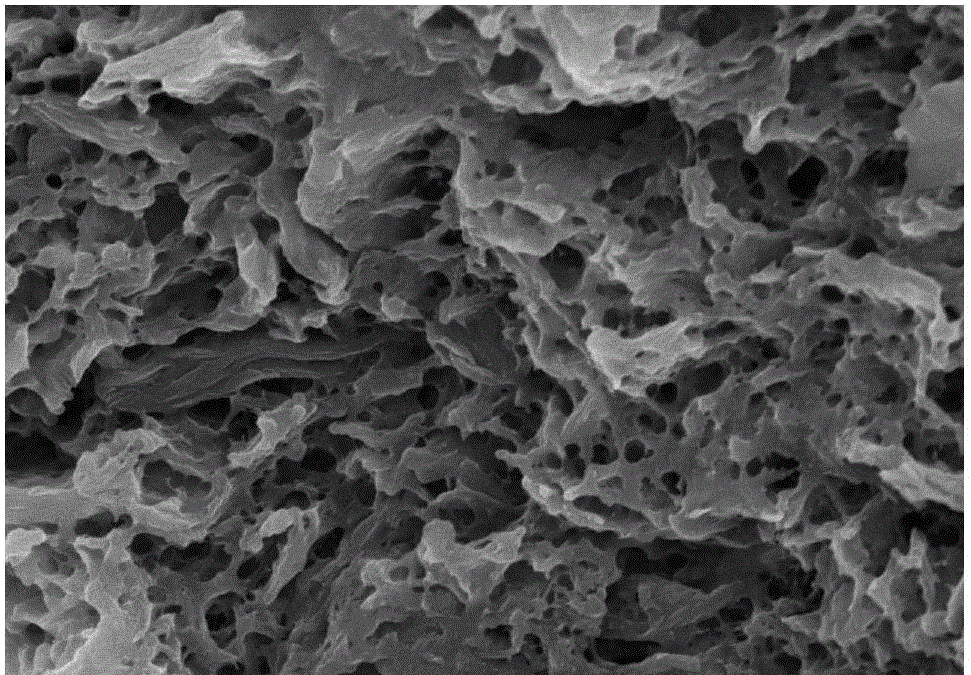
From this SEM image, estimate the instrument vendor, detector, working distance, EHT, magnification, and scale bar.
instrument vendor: Hitachi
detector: SE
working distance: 10 mm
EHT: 15 kV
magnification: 8 K X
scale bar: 5000 nm